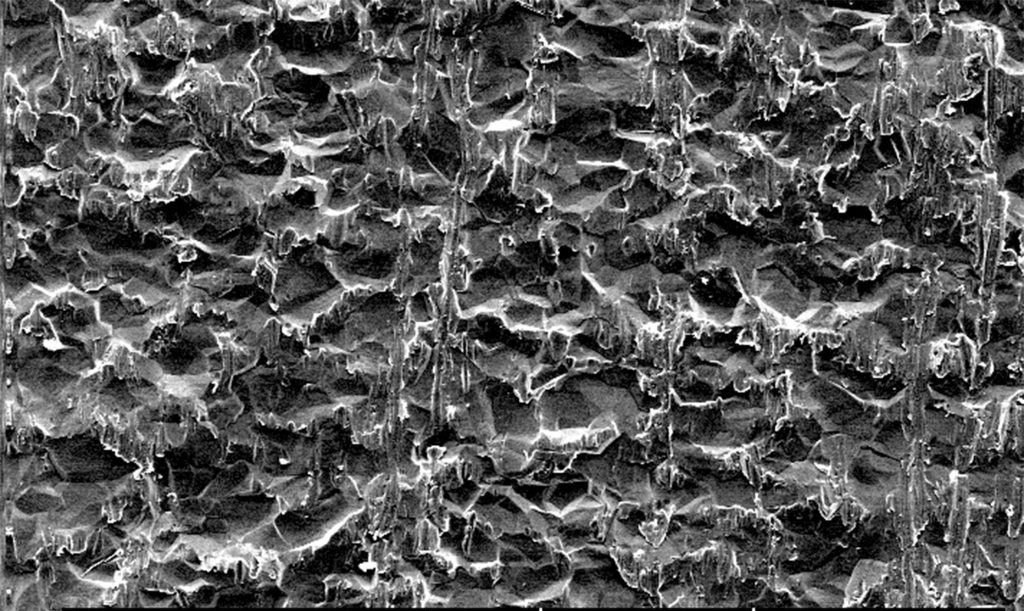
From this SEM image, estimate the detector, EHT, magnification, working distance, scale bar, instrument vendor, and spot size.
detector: SE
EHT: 10 kV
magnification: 0.5 K X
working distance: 10.1 mm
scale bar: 50000 nm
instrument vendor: FEI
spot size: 4